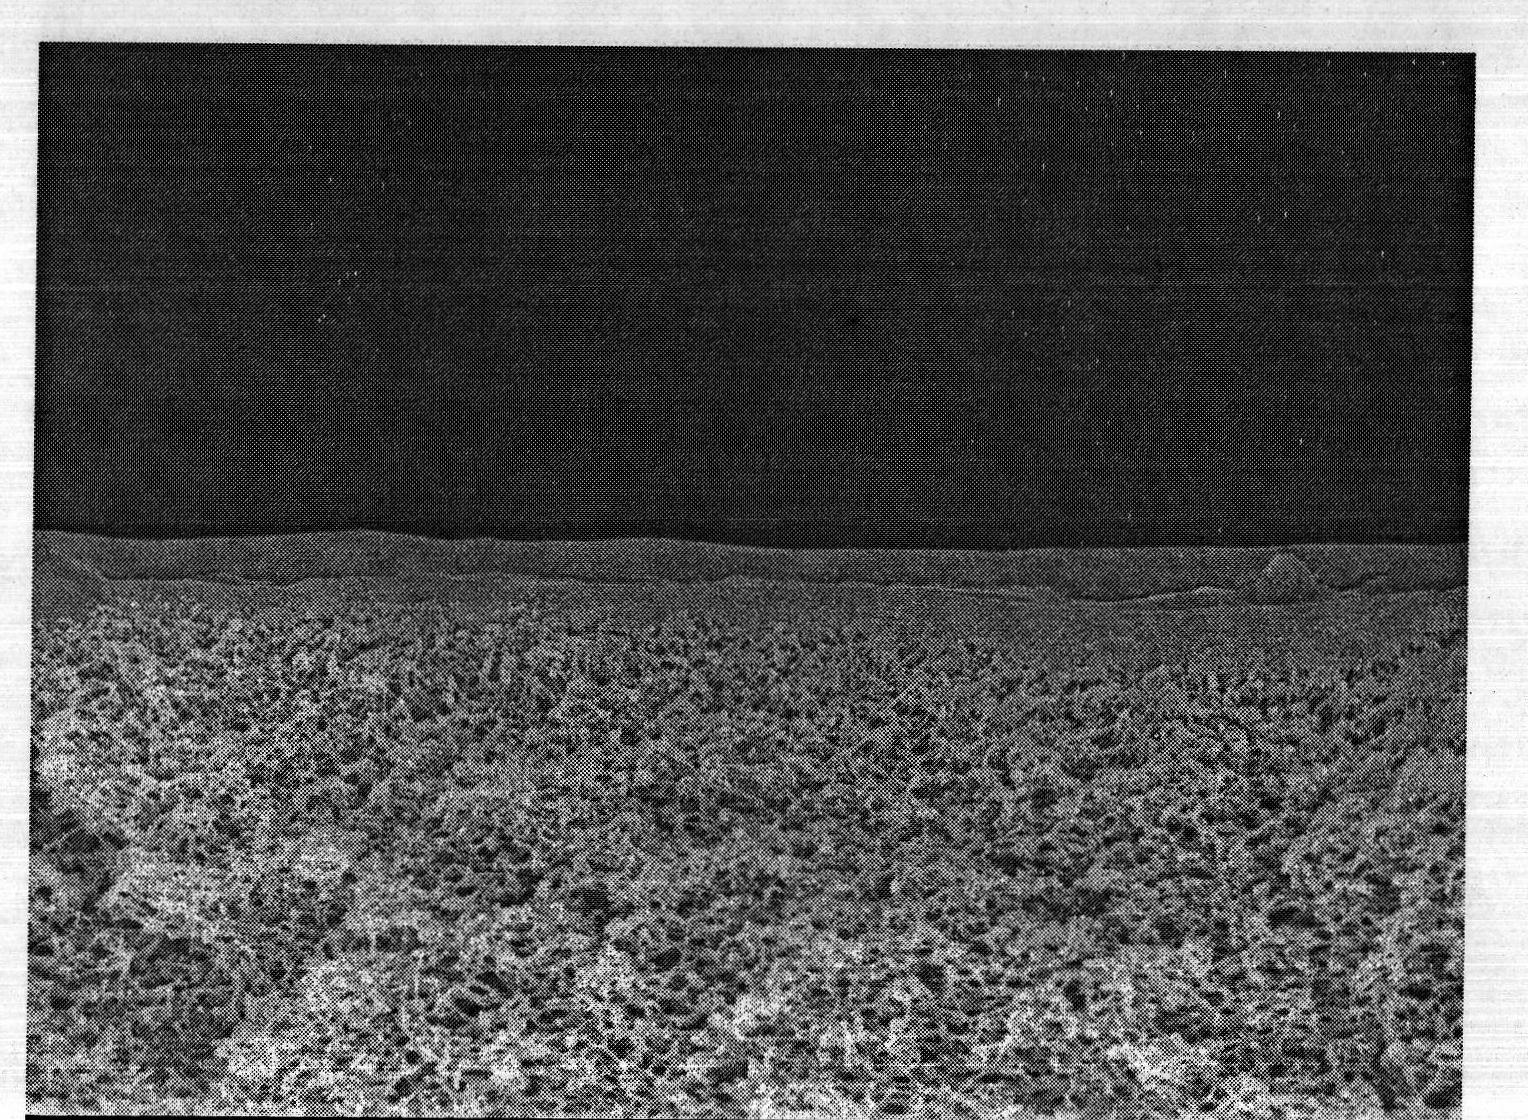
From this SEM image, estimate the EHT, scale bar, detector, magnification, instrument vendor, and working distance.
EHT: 10 kV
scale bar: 1000 nm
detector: SEI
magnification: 5 K X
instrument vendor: JEOL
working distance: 8.3 mm